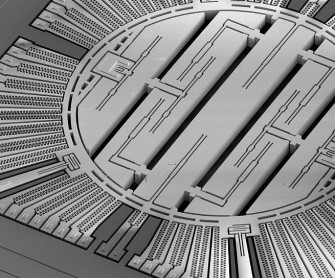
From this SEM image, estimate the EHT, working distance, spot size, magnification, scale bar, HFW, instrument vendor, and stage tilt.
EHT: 2 kV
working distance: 10.1 mm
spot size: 3.5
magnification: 0.175 K X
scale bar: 300000 nm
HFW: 853 µm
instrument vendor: FEI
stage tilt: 45°